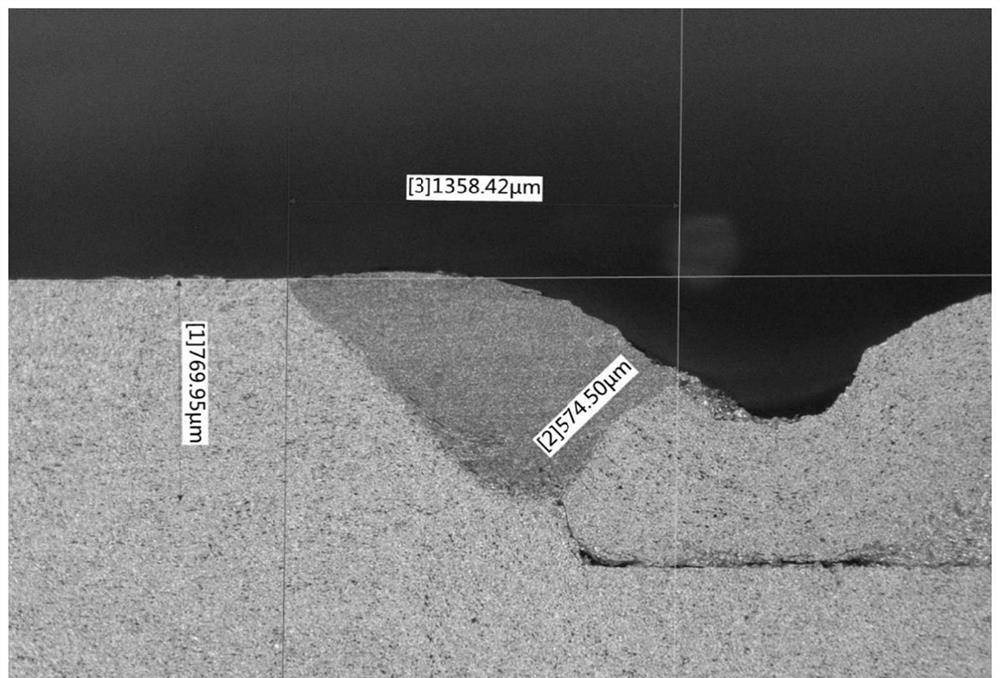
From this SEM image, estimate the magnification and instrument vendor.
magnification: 0.1 K X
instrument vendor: other